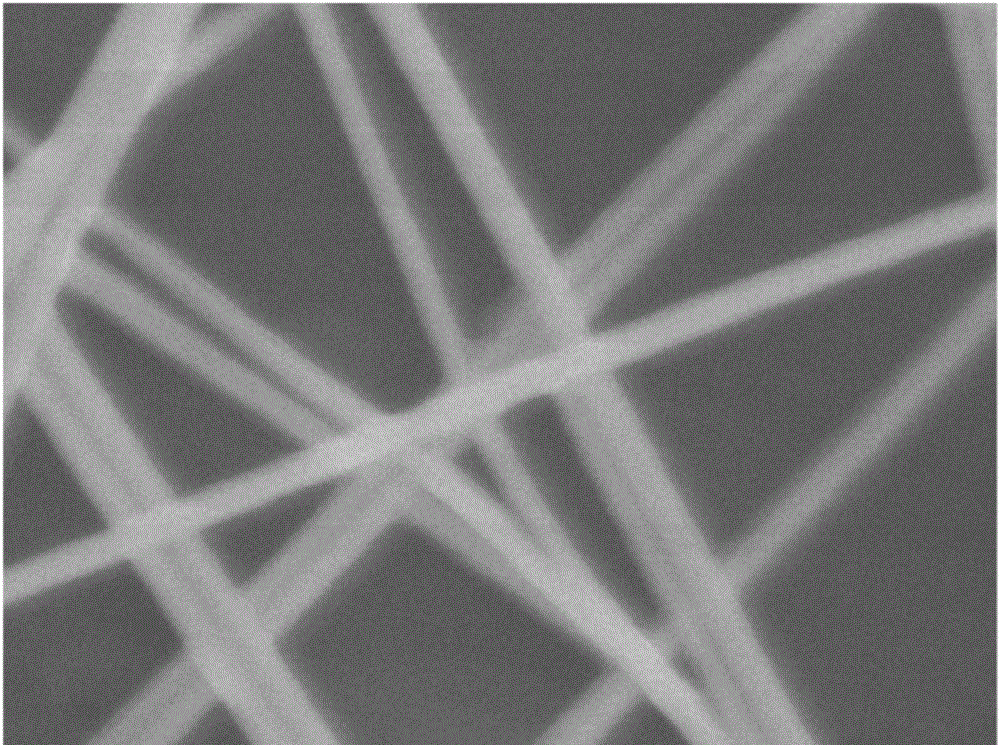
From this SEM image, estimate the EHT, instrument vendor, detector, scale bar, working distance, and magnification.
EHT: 5 kV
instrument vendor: JEOL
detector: SEI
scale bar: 100 nm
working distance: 8.4 mm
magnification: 60 K X